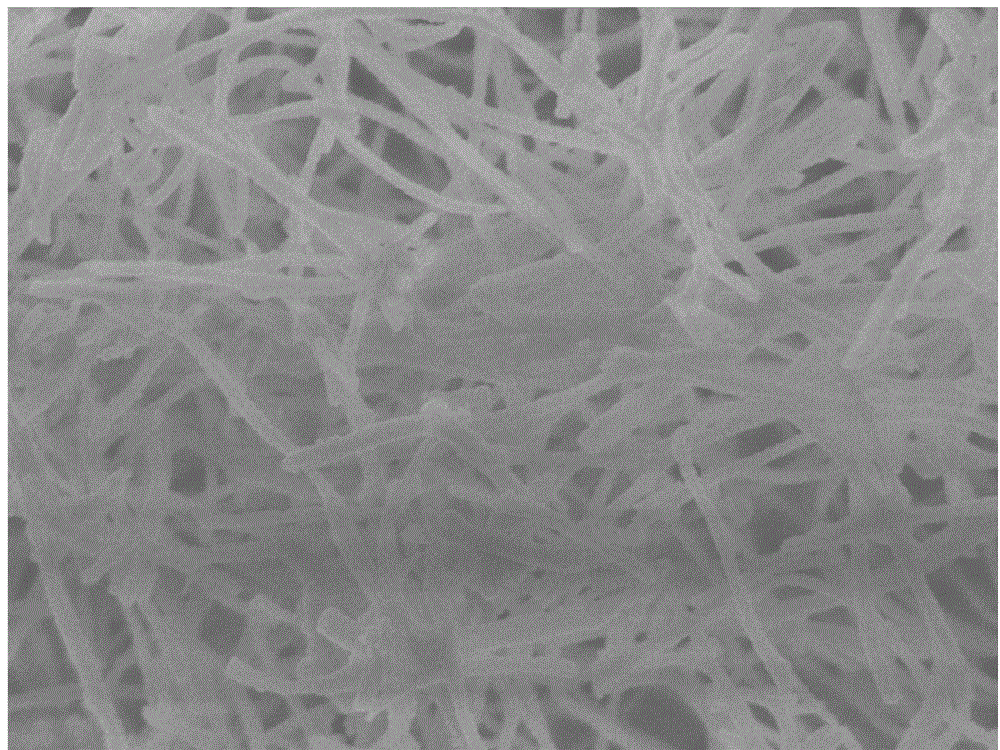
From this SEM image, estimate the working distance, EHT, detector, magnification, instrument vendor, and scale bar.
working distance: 6.5 mm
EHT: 3 kV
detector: SEI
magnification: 20 K X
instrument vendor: JEOL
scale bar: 1000 nm